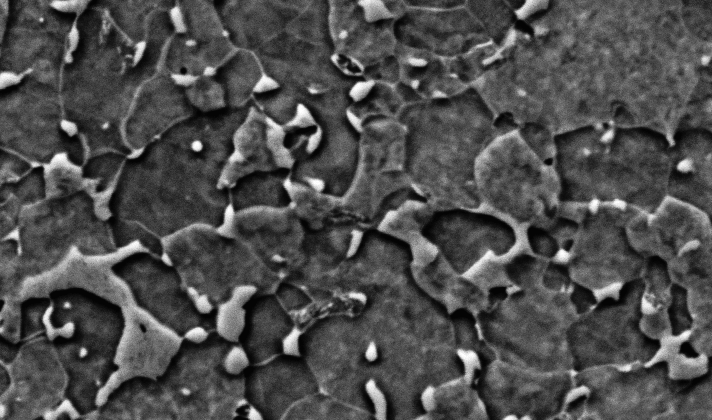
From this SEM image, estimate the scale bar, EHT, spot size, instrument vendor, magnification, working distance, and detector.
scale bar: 10000 nm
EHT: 20 kV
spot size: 5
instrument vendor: FEI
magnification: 3.5 K X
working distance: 11 mm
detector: BSE